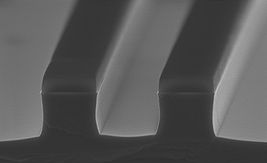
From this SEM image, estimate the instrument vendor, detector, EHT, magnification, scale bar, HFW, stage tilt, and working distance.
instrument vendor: Zeiss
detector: InLens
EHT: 5 kV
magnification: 65.74 K X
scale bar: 200 nm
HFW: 4.579 µm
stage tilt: -6.2°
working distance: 2.9 mm